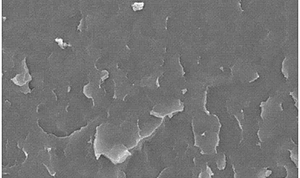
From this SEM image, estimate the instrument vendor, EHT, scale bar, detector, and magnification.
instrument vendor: JEOL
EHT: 20 kV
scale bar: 10000 nm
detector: SEI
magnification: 1 K X